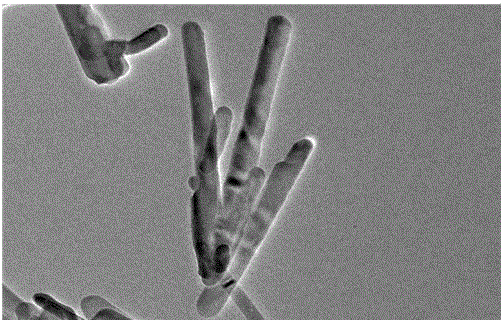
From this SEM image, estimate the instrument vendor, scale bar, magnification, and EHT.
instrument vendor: JEOL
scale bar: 100 nm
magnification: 40 K X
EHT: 200 kV